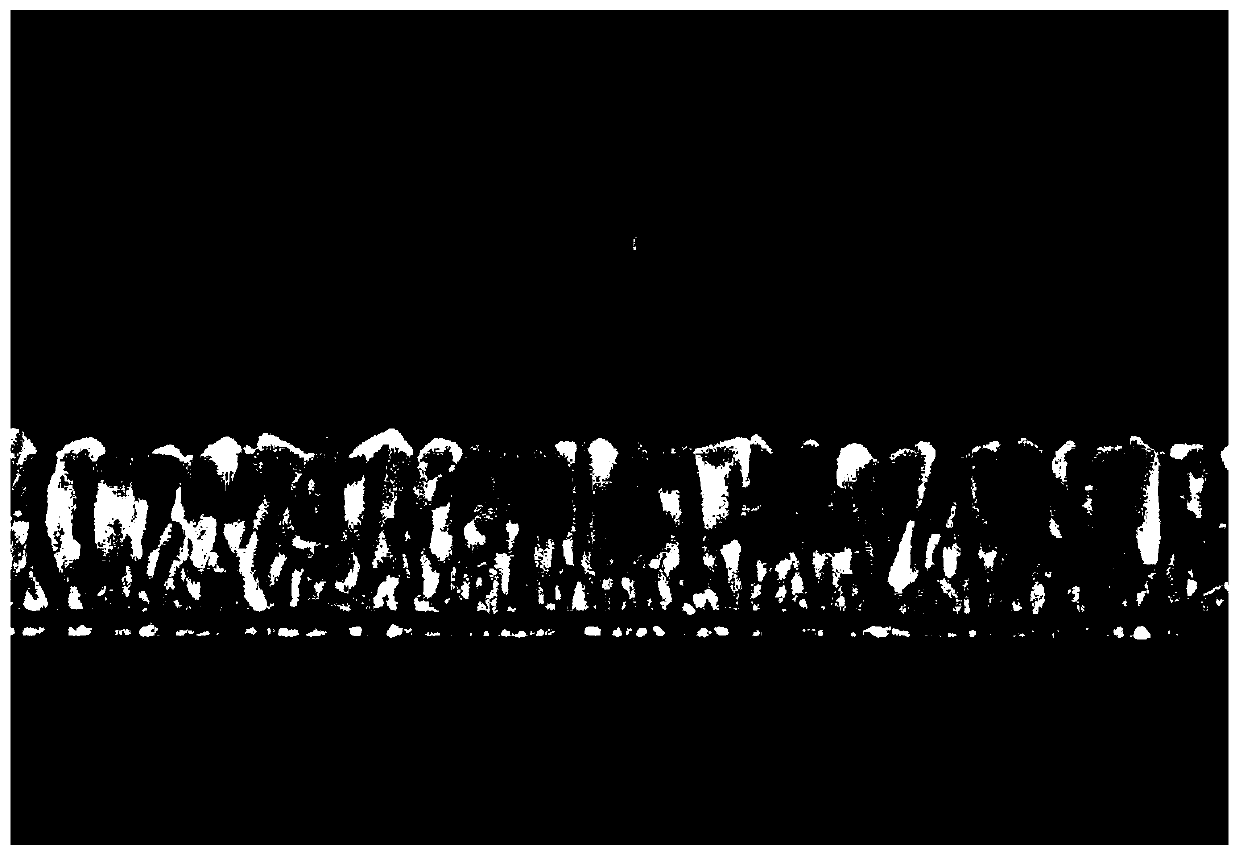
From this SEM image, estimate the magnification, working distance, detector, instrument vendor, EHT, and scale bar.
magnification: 50 K X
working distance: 6.7 mm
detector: SE(U)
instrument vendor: Hitachi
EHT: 3 kV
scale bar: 1000 nm